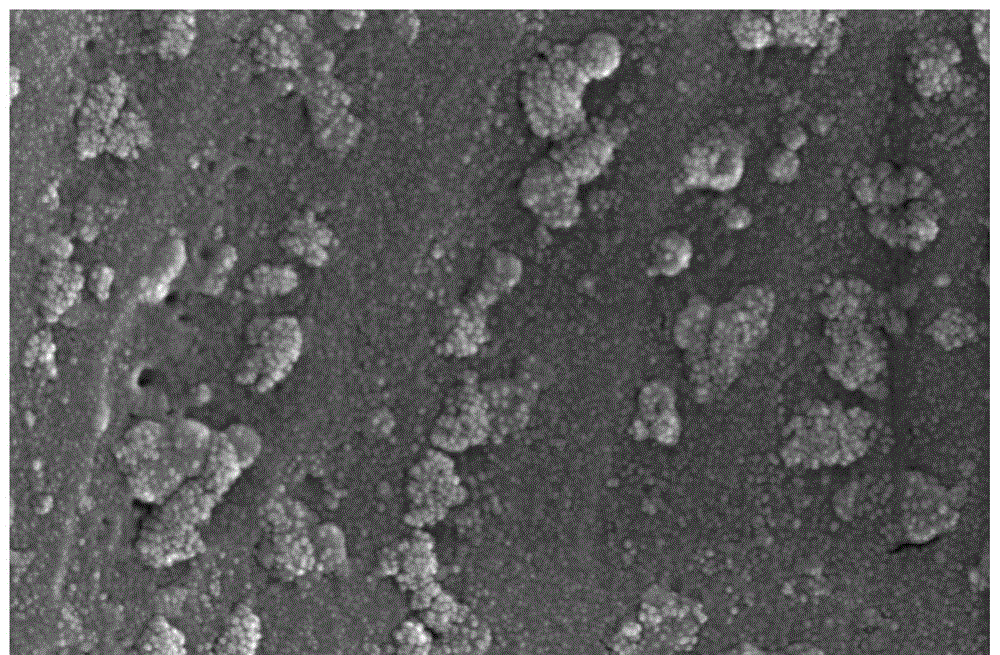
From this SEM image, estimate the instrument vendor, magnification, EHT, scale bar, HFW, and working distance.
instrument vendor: FEI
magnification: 150 K X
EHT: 25 kV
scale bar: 1000 nm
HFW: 2.76 µm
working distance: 10 mm